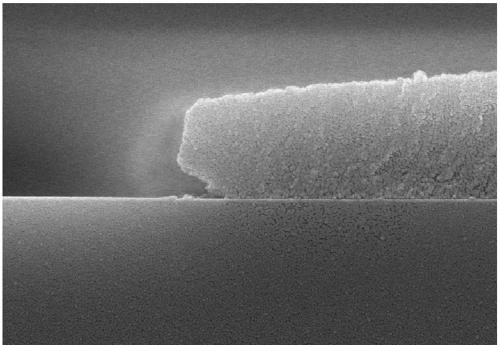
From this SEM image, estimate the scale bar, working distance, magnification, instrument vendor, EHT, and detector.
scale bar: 2000 nm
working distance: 7.9 mm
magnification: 20 K X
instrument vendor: Hitachi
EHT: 15 kV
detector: SE(UL)